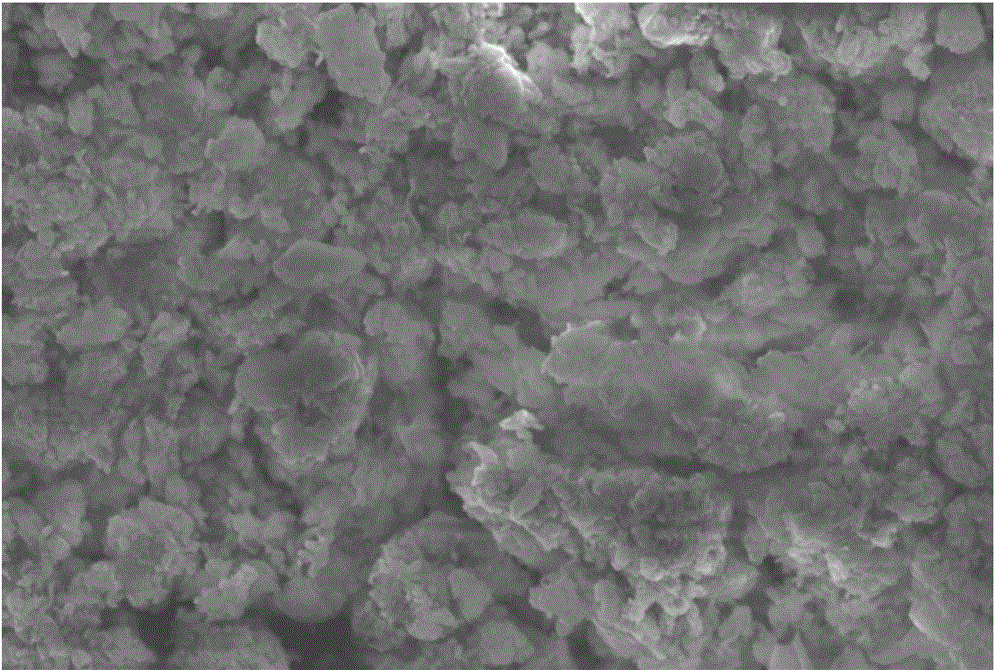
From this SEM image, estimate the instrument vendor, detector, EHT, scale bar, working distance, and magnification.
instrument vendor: Hitachi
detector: SE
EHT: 15 kV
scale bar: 20000 nm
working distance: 18.4 mm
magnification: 1.5 K X